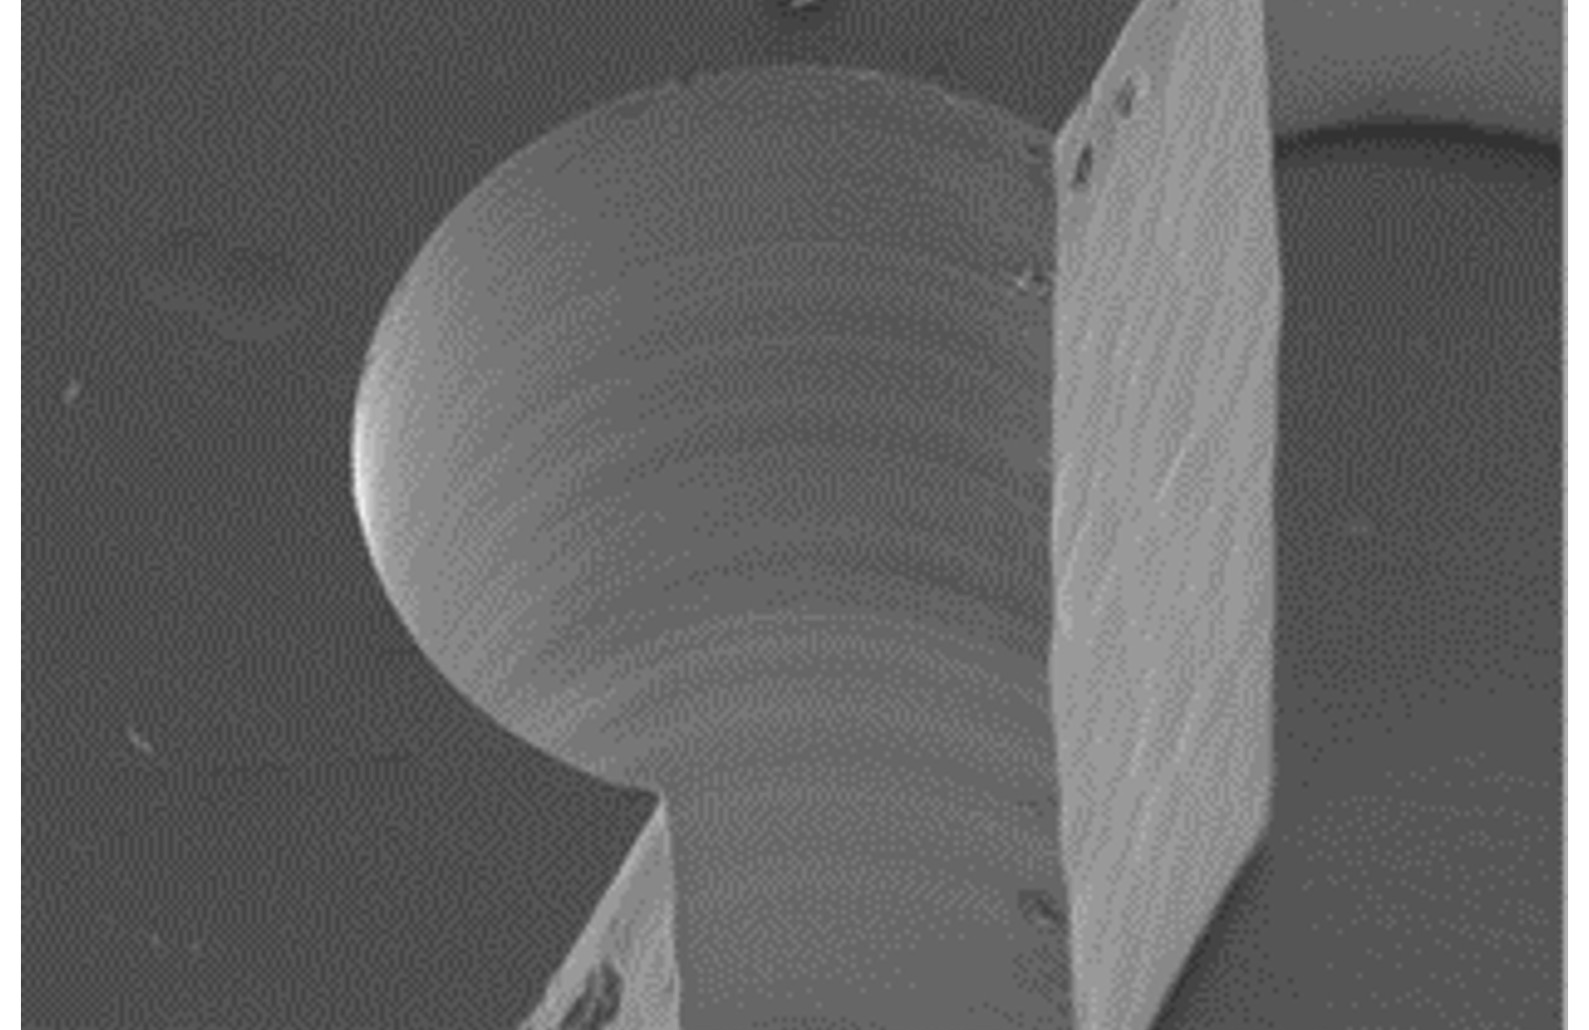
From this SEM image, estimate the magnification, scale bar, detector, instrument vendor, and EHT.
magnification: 0.21 K X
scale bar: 200000 nm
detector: SE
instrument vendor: Hitachi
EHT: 15 kV